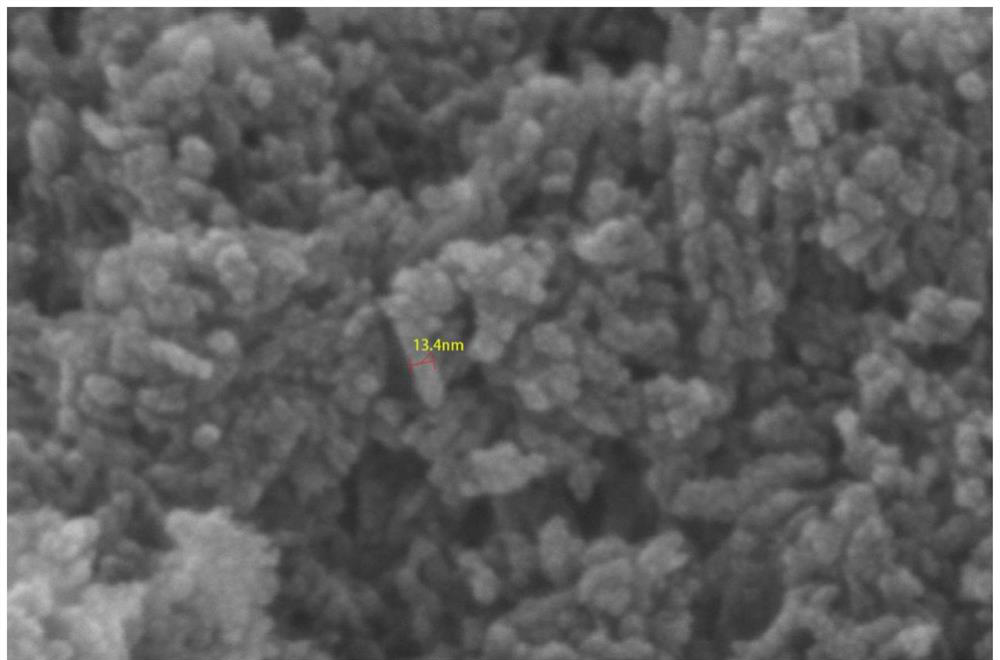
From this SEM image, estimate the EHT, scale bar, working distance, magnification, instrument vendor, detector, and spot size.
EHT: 15 kV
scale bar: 200 nm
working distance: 5.9 mm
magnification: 800 K X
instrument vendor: FEI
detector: TLD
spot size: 3